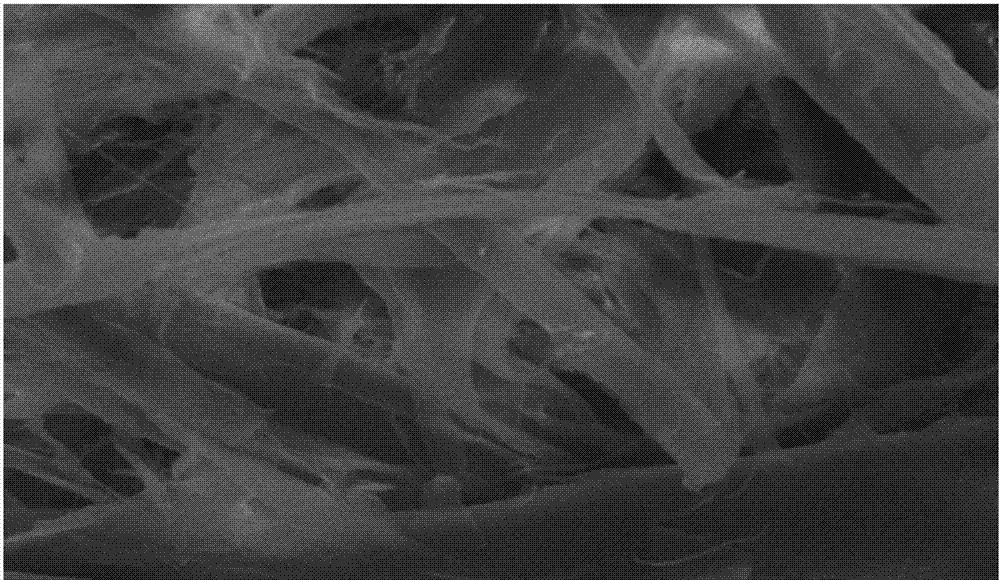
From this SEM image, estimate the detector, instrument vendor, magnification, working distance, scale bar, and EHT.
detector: SE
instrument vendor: Hitachi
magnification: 1 K X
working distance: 10.9 mm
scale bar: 50000 nm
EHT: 20 kV